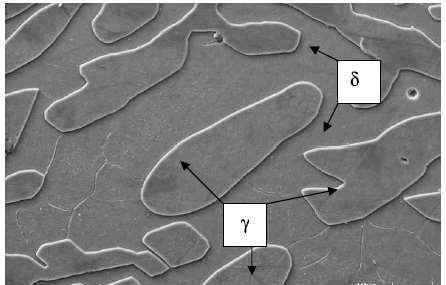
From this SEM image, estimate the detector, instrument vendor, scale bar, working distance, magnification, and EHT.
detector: SE1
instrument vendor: Zeiss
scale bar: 10000 nm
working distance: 25 mm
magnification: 3 K X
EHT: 20 kV